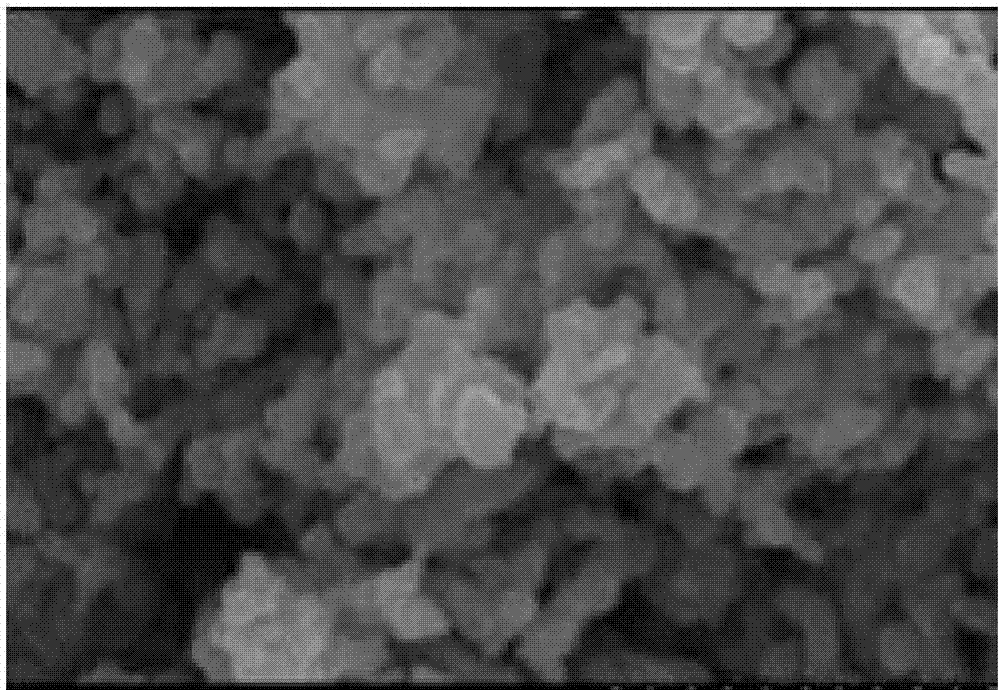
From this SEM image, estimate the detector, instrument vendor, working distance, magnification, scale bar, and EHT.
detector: SE(M)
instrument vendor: Hitachi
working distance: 13.7 mm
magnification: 10 K X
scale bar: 5000 nm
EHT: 10 kV